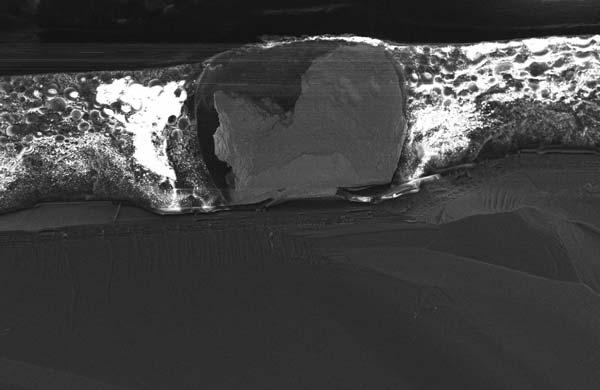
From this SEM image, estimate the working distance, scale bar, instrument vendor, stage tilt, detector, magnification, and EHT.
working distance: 4 mm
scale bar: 100000 nm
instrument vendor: Zeiss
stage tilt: -15°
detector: InLens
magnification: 0.678 K X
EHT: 15 kV